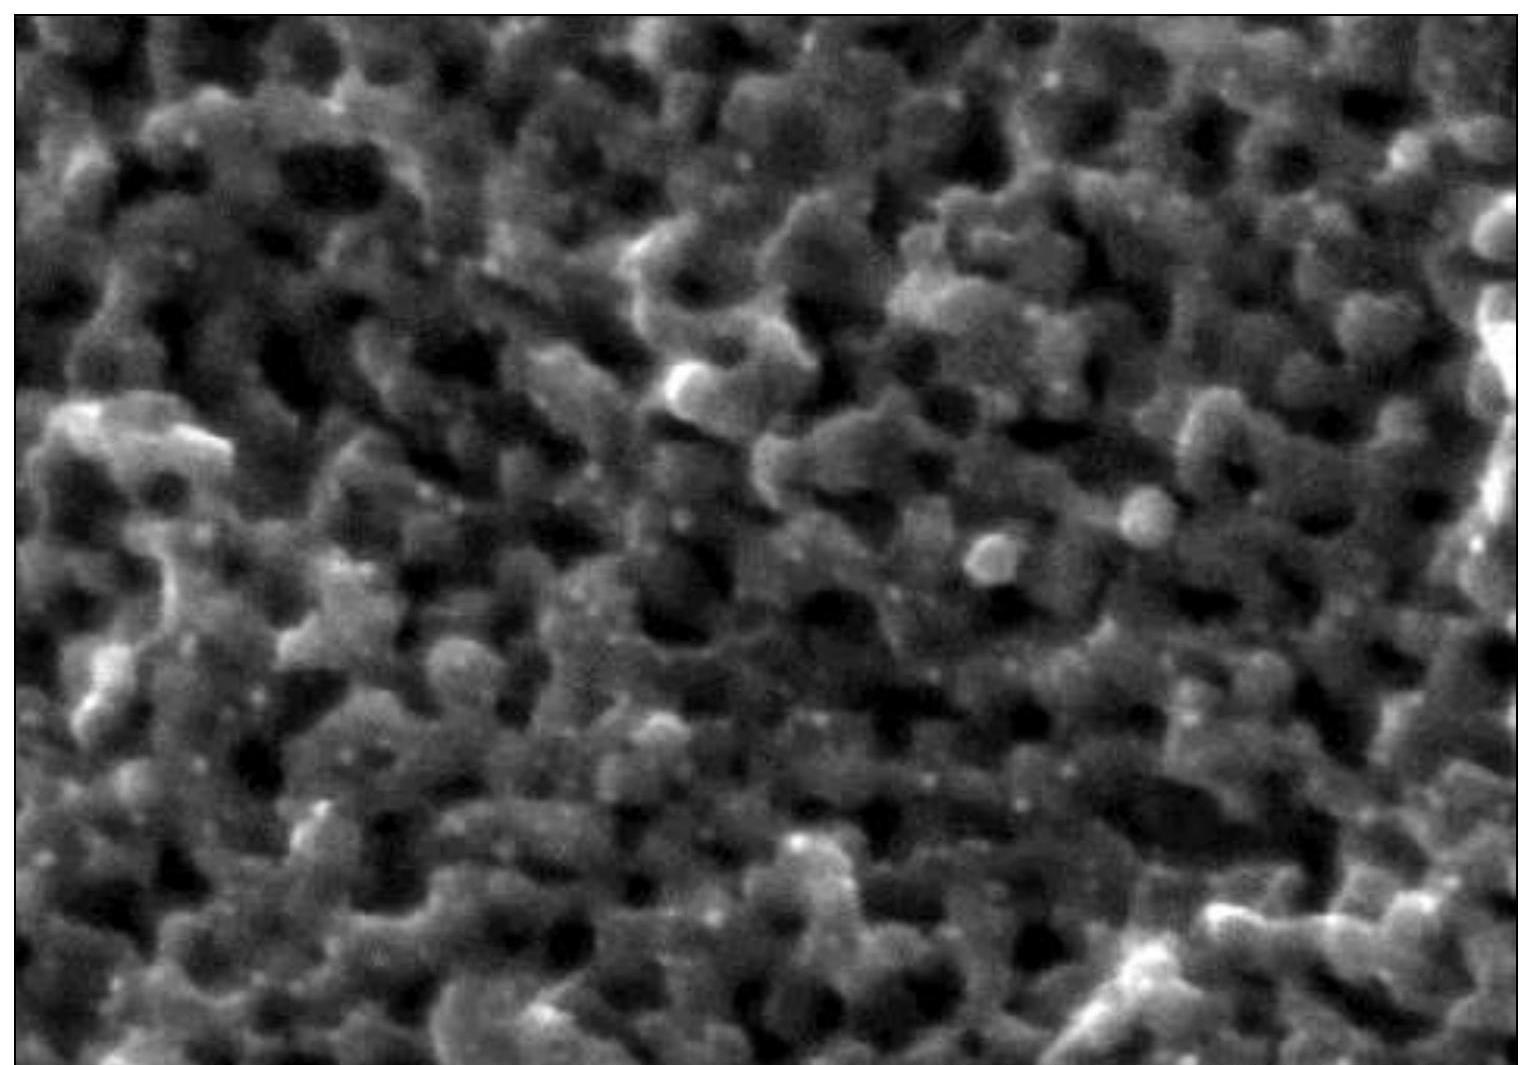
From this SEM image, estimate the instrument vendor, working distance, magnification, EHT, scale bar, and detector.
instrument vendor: Hitachi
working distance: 7.5 mm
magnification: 450 K X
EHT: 10 kV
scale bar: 100 nm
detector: SE(UL)